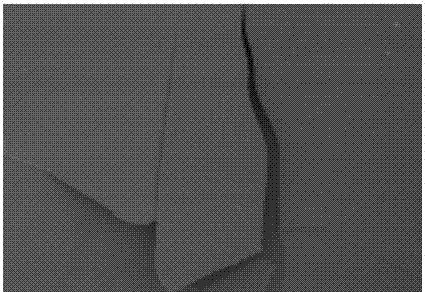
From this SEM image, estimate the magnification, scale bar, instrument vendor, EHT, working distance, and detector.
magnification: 2 K X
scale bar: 20000 nm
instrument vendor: Hitachi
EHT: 2 kV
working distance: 10.6 mm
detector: SE(M)